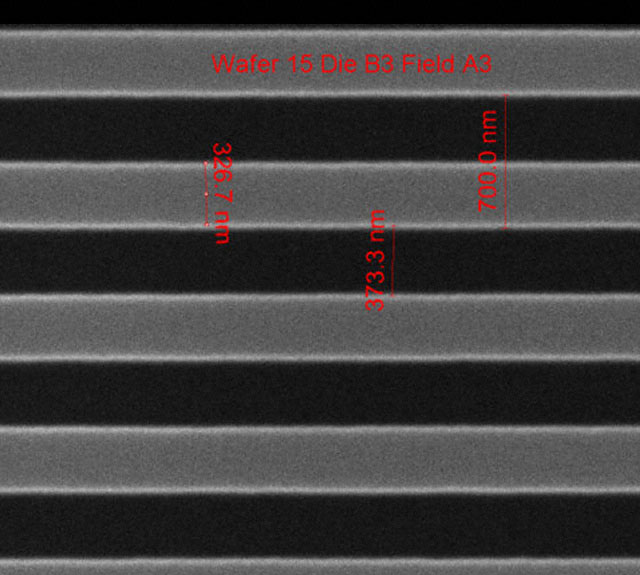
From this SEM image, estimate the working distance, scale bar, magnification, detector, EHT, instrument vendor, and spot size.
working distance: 10.2 mm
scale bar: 1000 nm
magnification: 75.019 K X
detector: ETD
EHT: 10 kV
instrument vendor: FEI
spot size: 1.5